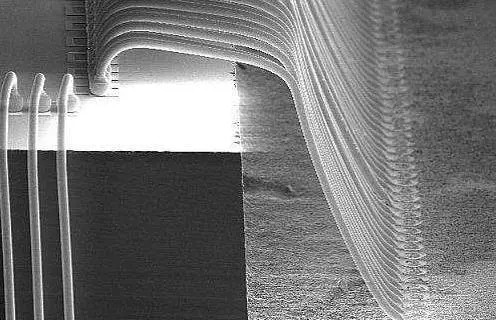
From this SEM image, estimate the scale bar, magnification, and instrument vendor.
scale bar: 200000 nm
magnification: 0.1 K X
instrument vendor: other